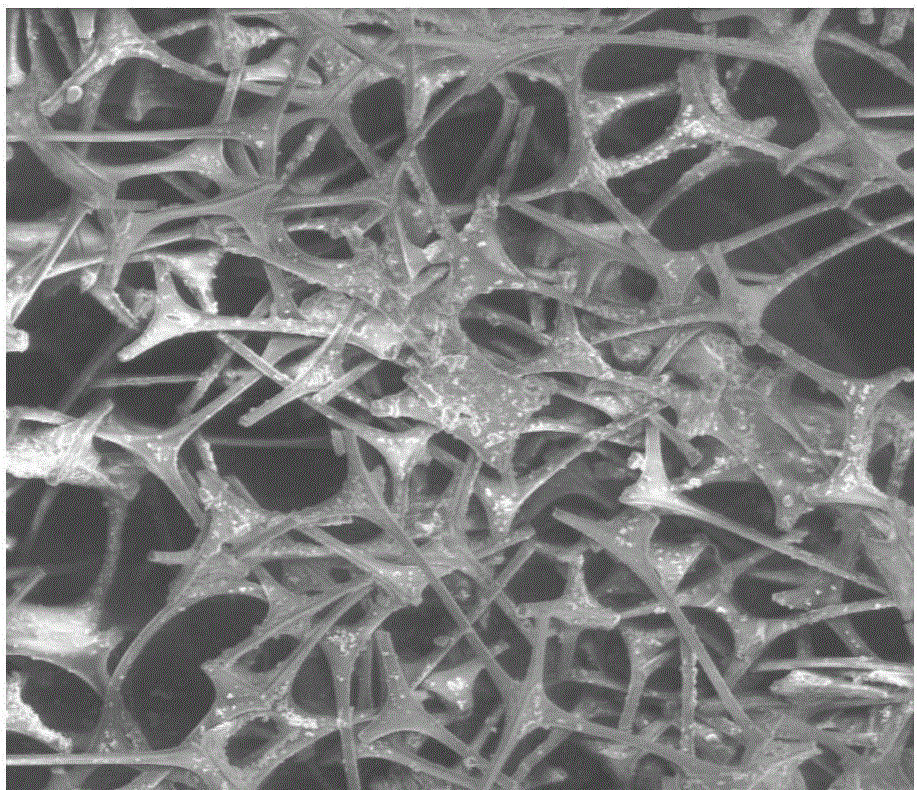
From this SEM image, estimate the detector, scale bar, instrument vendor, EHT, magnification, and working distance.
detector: LFD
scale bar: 200000 nm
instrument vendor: FEI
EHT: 10 kV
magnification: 0.7 K X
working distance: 10.1 mm